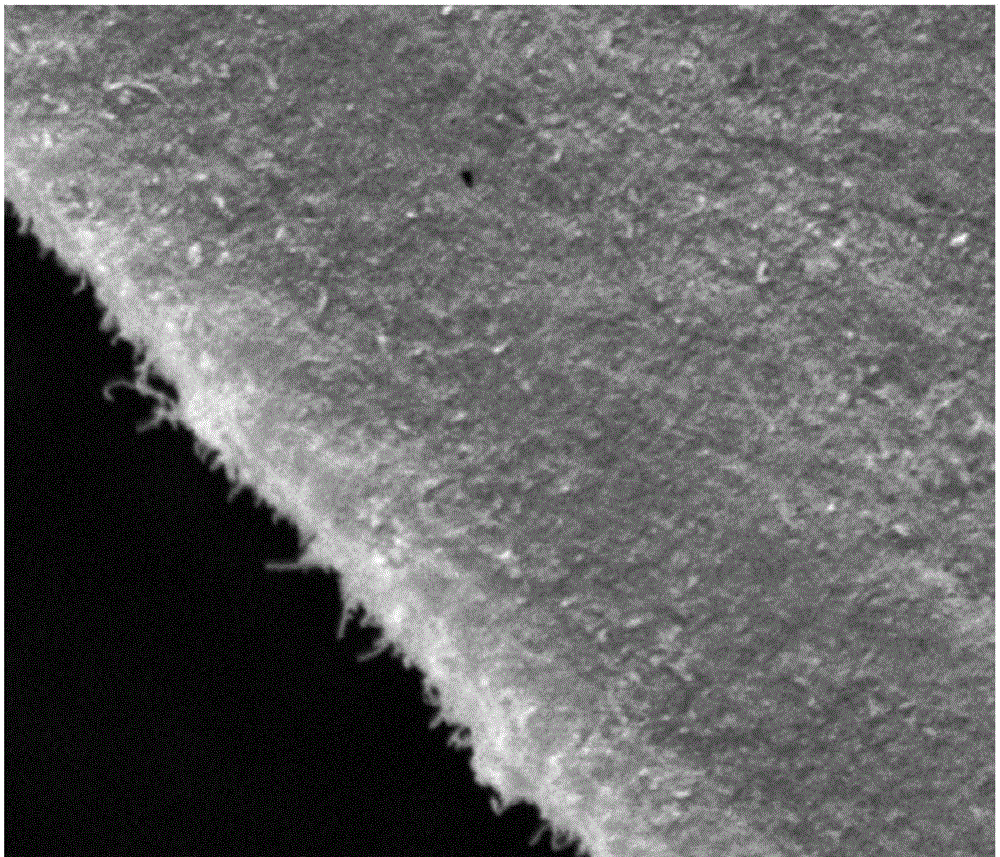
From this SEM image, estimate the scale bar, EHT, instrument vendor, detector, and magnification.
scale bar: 5000 nm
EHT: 12.5 kV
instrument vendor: FEI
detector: ETD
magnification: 10 K X